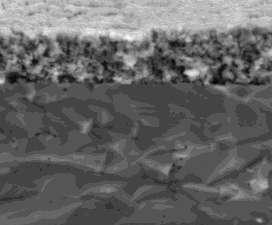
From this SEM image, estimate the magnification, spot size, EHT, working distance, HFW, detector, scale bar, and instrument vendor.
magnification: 10 K X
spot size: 4.5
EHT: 18 kV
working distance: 5.7 mm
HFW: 29.8 µm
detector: LVD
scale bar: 10000 nm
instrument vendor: FEI